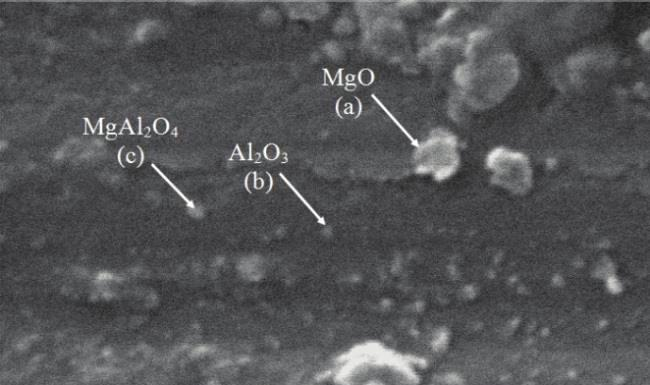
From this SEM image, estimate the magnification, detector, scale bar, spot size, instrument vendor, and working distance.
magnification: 32 K X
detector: SE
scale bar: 500 nm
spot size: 2.2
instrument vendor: FEI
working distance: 6.4 mm